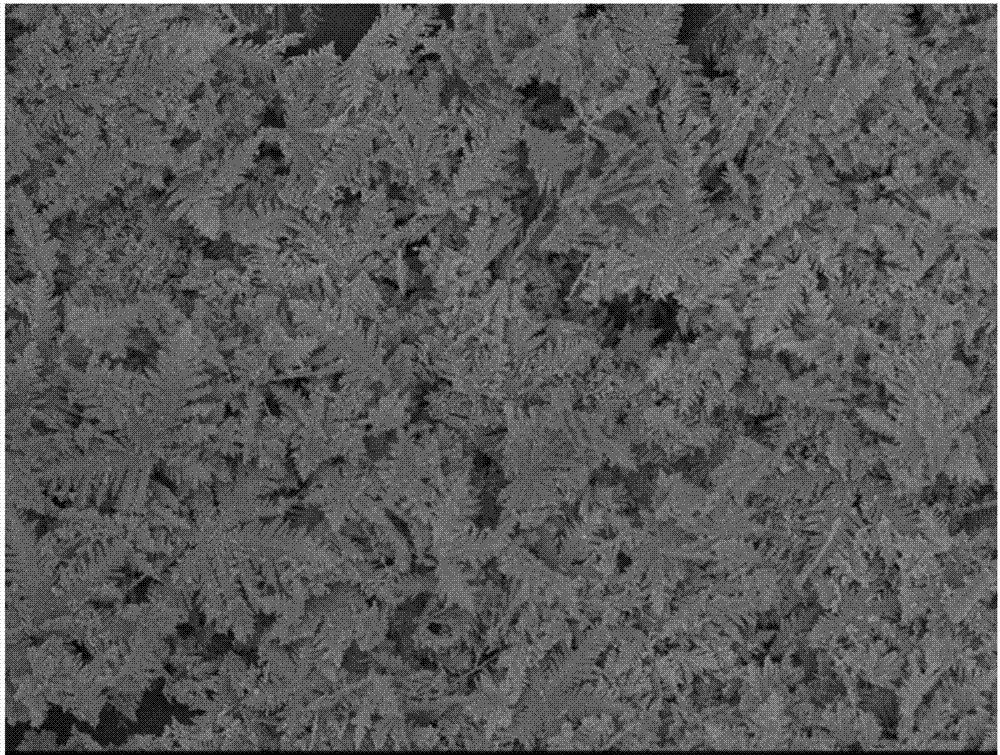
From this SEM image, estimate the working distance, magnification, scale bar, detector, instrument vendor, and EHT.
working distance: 6.1 mm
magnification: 2 K X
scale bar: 10000 nm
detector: SEI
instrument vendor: JEOL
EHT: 5 kV